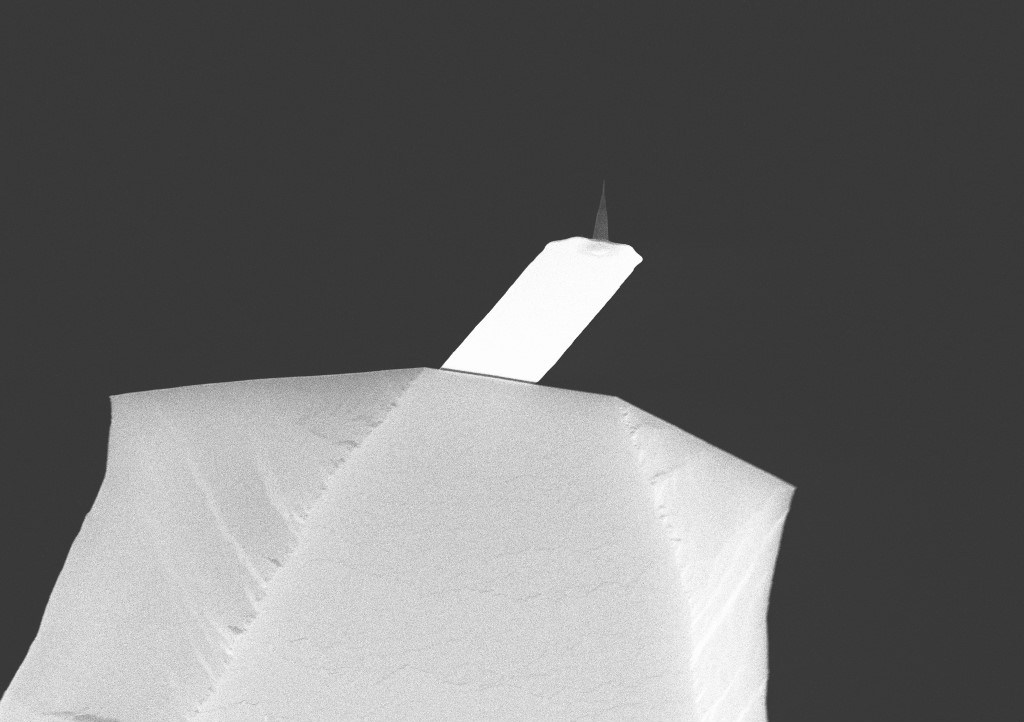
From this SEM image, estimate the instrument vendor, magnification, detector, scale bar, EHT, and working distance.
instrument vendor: Zeiss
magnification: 7.02 K X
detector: InLens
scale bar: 2000 nm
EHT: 20 kV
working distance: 7.9 mm